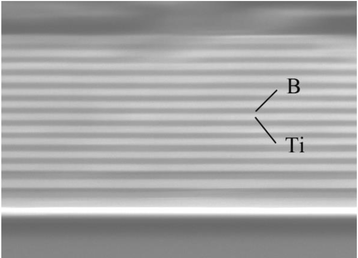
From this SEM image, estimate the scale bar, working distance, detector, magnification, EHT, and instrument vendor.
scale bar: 1000 nm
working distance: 7.8 mm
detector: SEI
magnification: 10 K X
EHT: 15 kV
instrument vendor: JEOL